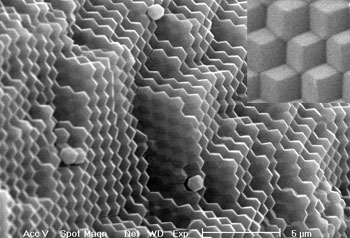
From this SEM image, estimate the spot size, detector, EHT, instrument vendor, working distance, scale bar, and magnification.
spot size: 4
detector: SE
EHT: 20 kV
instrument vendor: FEI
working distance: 5.3 mm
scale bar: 5000 nm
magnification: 5 K X